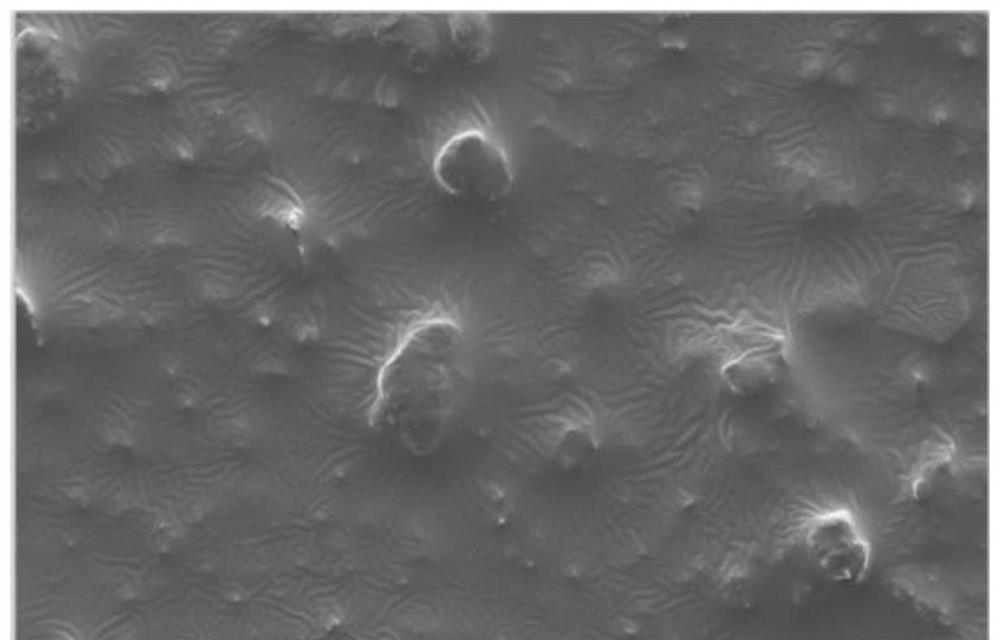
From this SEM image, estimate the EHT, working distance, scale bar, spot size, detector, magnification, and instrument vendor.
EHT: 5 kV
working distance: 19 mm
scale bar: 40000 nm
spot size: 3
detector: ETD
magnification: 5 K X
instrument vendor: FEI